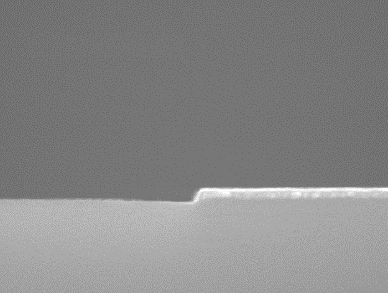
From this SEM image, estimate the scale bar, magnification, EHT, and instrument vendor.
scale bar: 300 nm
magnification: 100 K X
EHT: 10 kV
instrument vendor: Hitachi